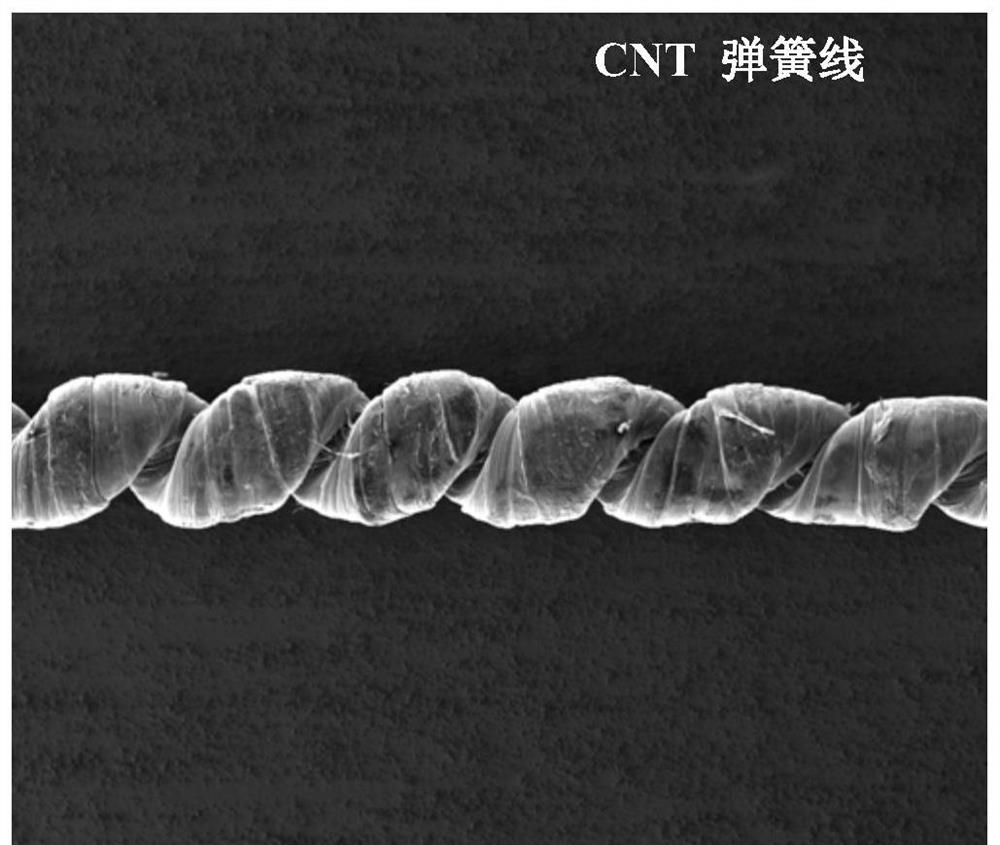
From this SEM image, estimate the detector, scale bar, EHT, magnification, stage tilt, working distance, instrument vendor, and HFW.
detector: ETD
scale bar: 500000 nm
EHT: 5 kV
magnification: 0.14 K X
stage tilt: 0°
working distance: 10.2 mm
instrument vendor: FEI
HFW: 2130 µm